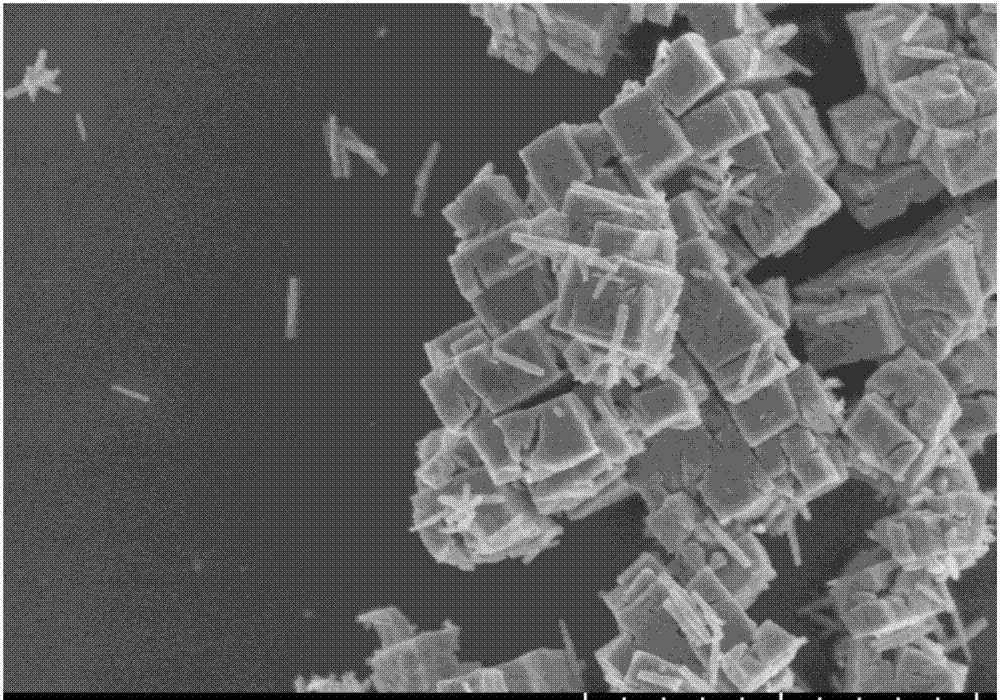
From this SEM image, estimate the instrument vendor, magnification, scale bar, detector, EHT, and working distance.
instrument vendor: Hitachi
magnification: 25 K X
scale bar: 2000 nm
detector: SE(UL)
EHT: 3 kV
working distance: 10 mm